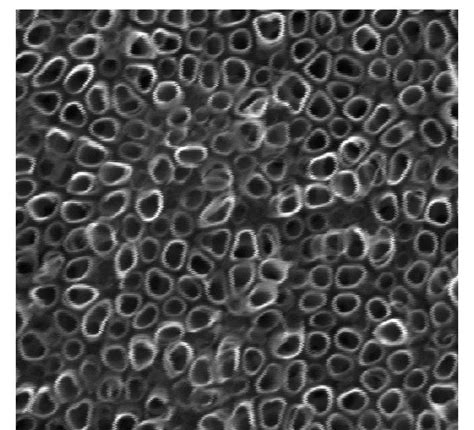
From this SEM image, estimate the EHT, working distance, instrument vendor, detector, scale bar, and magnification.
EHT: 20 kV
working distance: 10 mm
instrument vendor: FEI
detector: ETD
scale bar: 500 nm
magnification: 100 K X